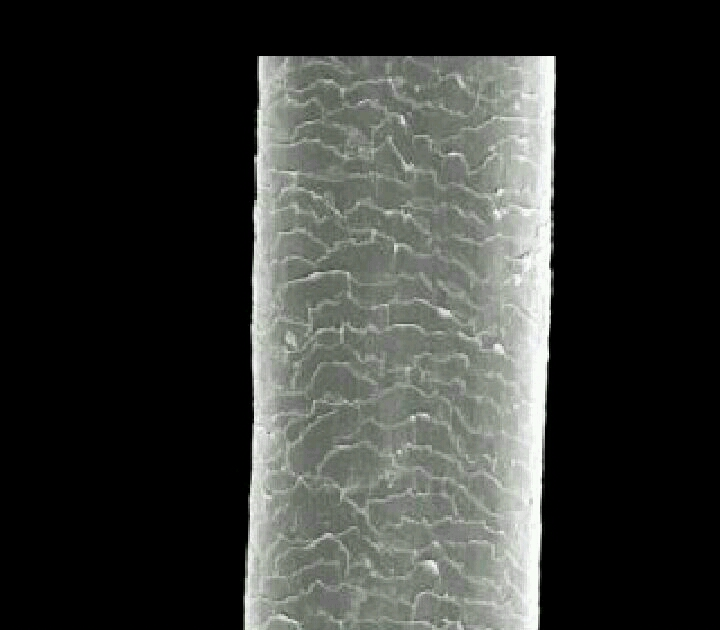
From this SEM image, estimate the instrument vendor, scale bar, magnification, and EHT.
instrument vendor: JEOL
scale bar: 50000 nm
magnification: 0.5 K X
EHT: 10 kV